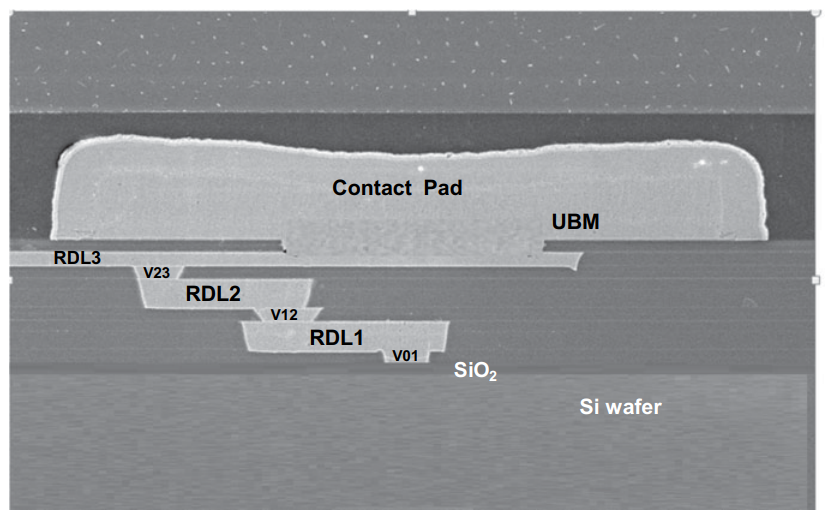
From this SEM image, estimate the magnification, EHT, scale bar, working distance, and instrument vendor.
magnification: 2 K X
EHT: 10 kV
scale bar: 20000 nm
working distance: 7 mm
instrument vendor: Hitachi